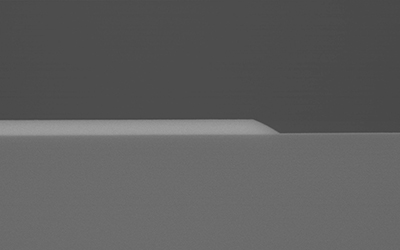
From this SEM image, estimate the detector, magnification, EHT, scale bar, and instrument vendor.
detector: YAGBSE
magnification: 30 K X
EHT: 10 kV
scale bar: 1000 nm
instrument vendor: Hitachi